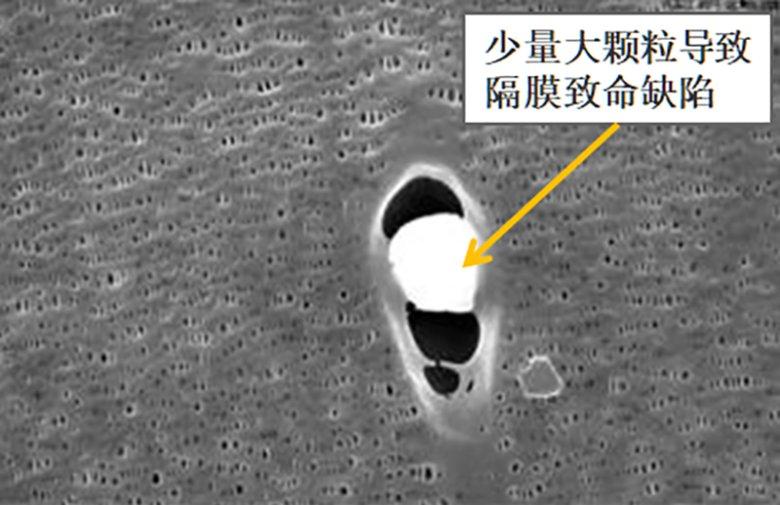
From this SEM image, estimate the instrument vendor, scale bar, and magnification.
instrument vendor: JEOL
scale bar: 1000 nm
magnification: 10 K X